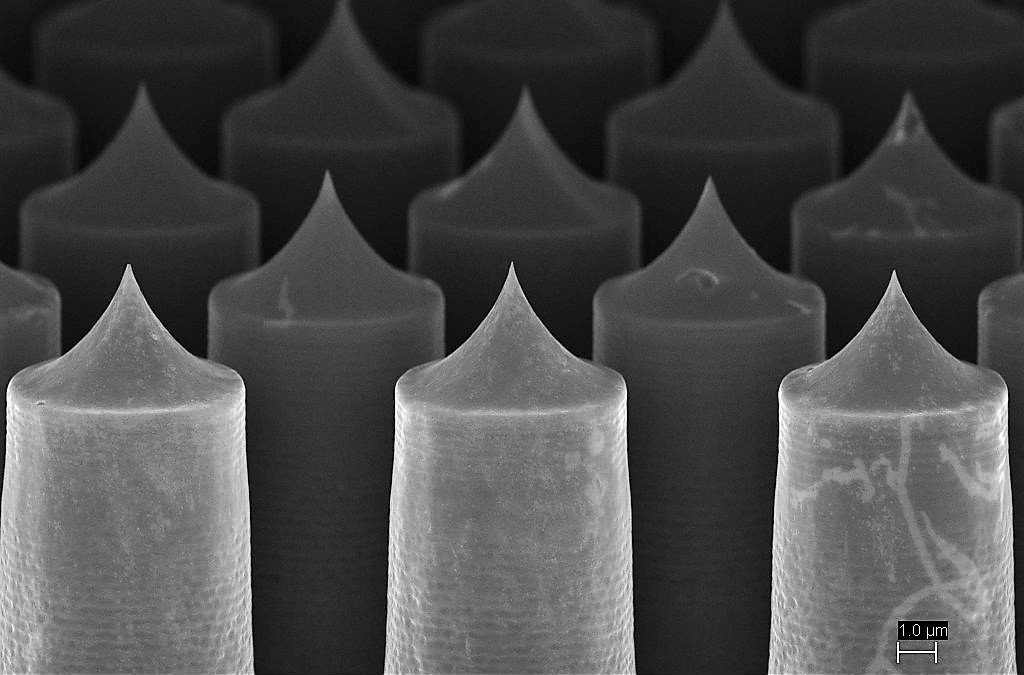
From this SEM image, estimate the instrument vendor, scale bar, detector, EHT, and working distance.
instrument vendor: Zeiss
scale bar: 2000 nm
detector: InLens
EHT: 5 kV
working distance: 5.5 mm